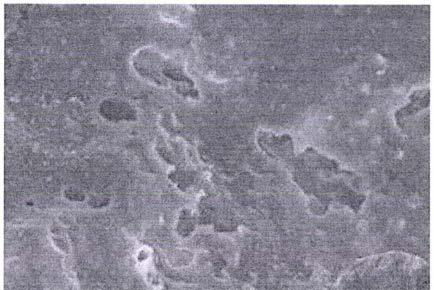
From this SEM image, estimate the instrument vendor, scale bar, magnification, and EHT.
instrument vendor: other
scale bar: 100000 nm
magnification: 0.5 K X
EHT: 25 kV